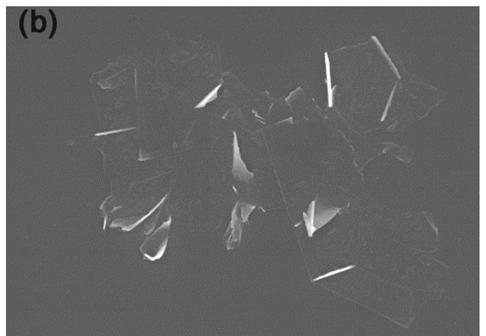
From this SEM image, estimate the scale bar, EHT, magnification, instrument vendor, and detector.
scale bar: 5000 nm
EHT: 10 kV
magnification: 10 K X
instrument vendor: Hitachi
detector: SE(M)